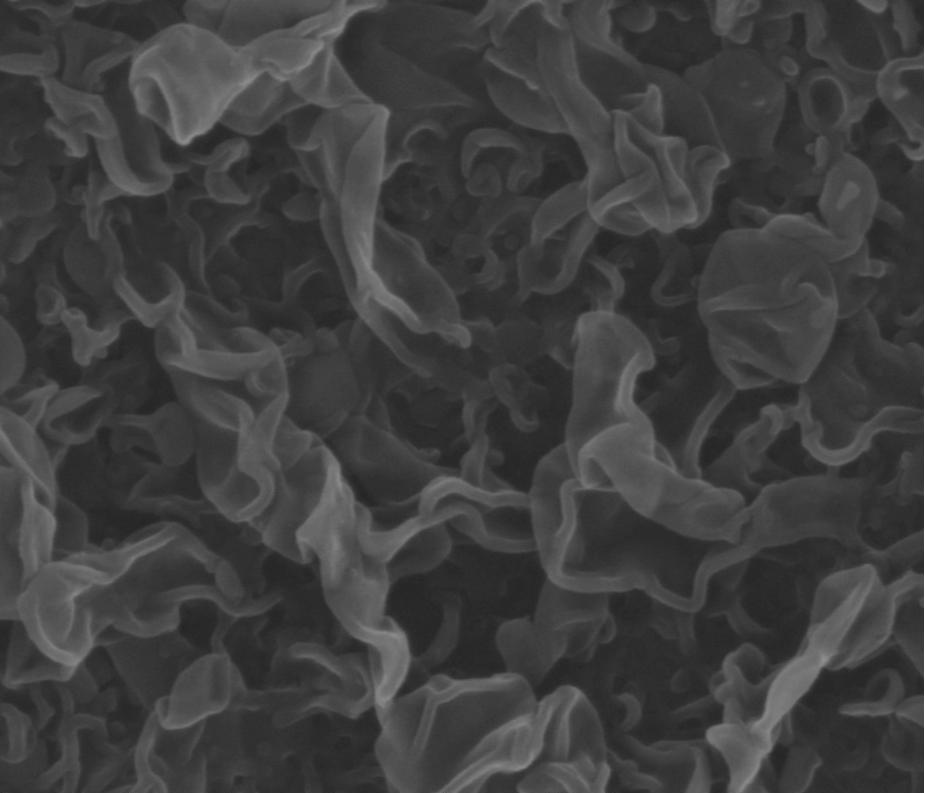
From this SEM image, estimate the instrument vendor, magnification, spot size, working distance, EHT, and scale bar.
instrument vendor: FEI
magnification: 100 K X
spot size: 2.5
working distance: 10 mm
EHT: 15 kV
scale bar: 500 nm